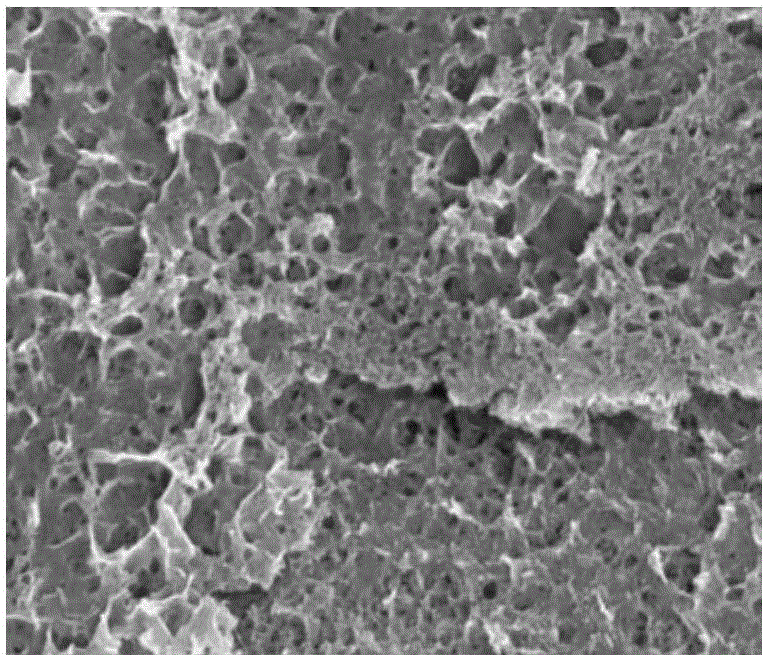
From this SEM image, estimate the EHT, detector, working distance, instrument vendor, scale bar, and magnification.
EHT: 10 kV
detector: TLD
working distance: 6.5 mm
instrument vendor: FEI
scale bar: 1000 nm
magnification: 80 K X